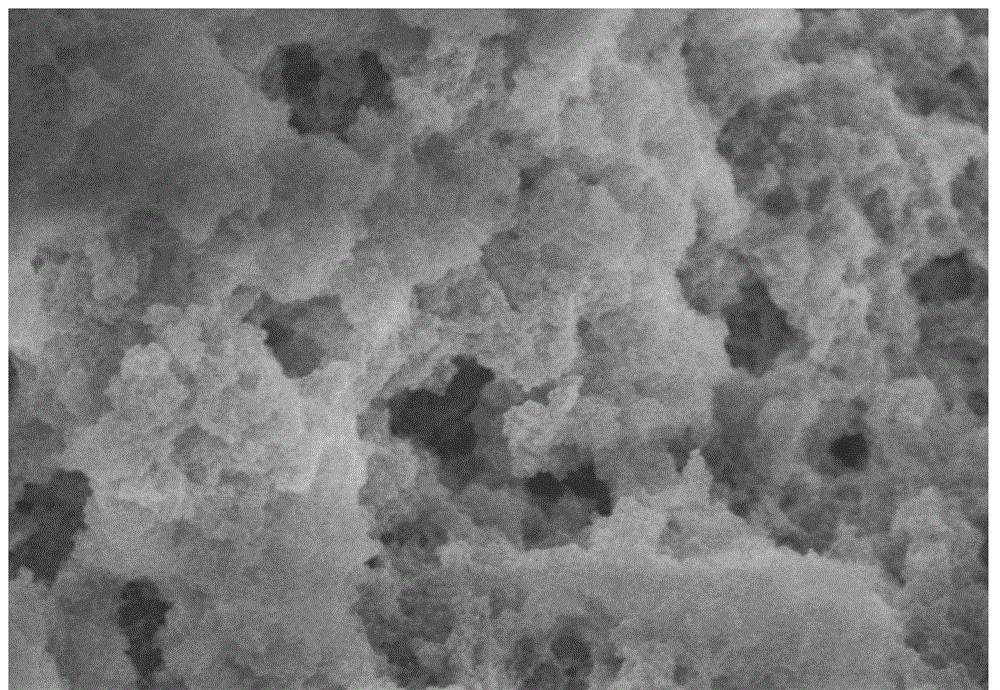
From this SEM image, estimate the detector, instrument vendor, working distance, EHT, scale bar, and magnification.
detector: SE(M)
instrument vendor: Hitachi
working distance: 9.6 mm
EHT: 5 kV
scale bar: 2000 nm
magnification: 25 K X